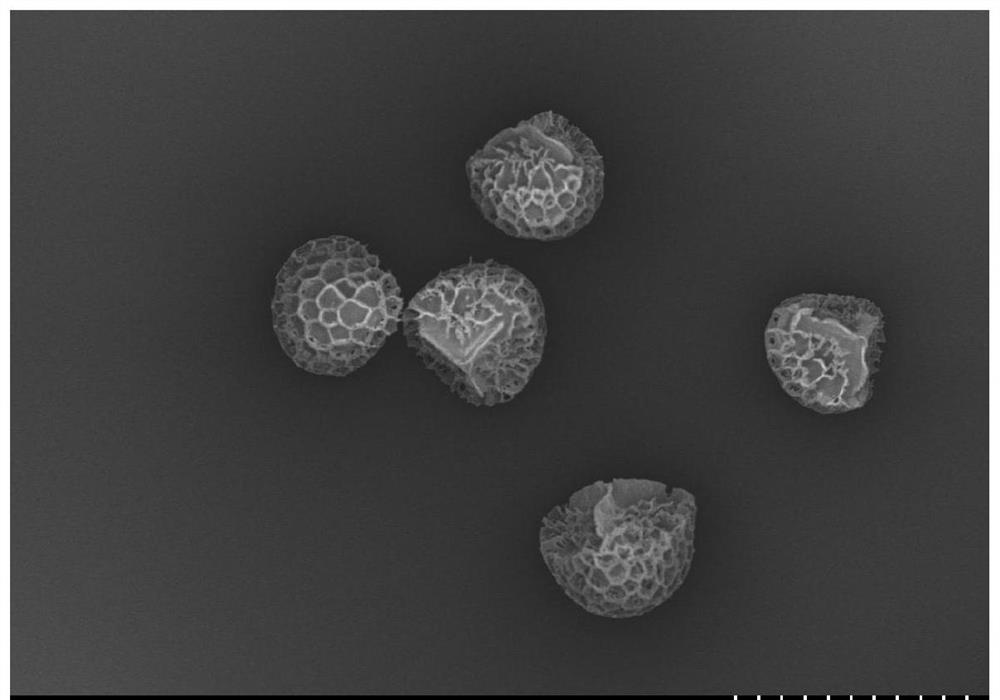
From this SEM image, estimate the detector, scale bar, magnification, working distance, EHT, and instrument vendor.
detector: SE(U)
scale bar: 50000 nm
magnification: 0.6 K X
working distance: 8.4 mm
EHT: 3 kV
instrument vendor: Hitachi